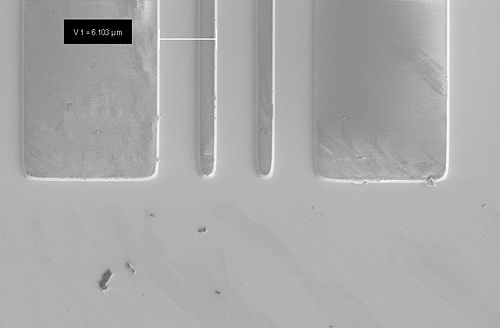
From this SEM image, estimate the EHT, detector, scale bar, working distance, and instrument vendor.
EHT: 2 kV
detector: SE2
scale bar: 2000 nm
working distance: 8.6 mm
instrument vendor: Zeiss